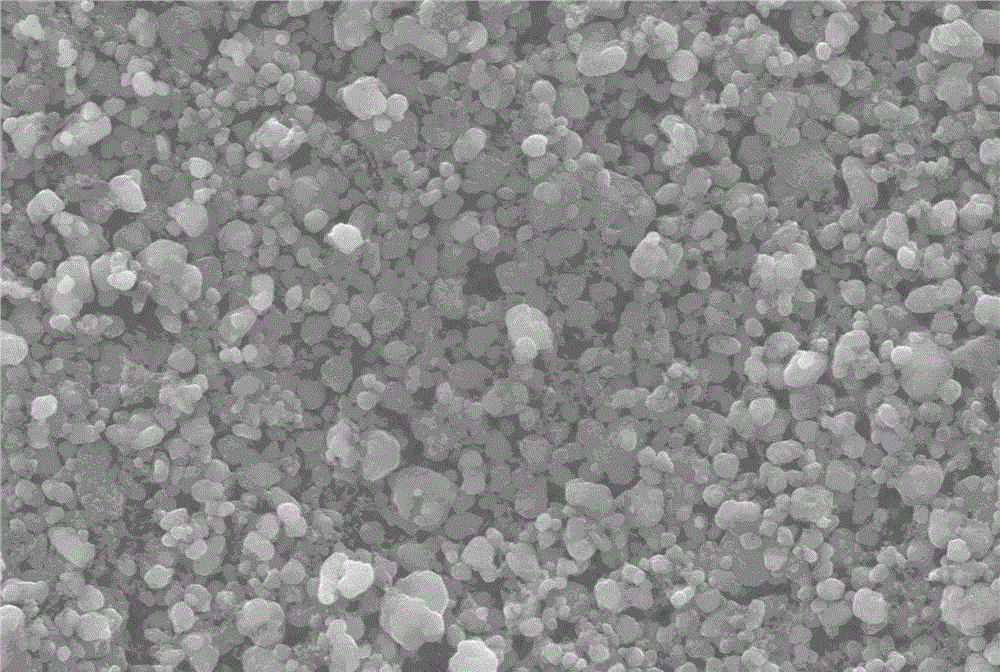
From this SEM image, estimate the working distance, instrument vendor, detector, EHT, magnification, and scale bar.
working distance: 7.5 mm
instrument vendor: Zeiss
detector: SE1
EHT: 15 kV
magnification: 5 K X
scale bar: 1000 nm